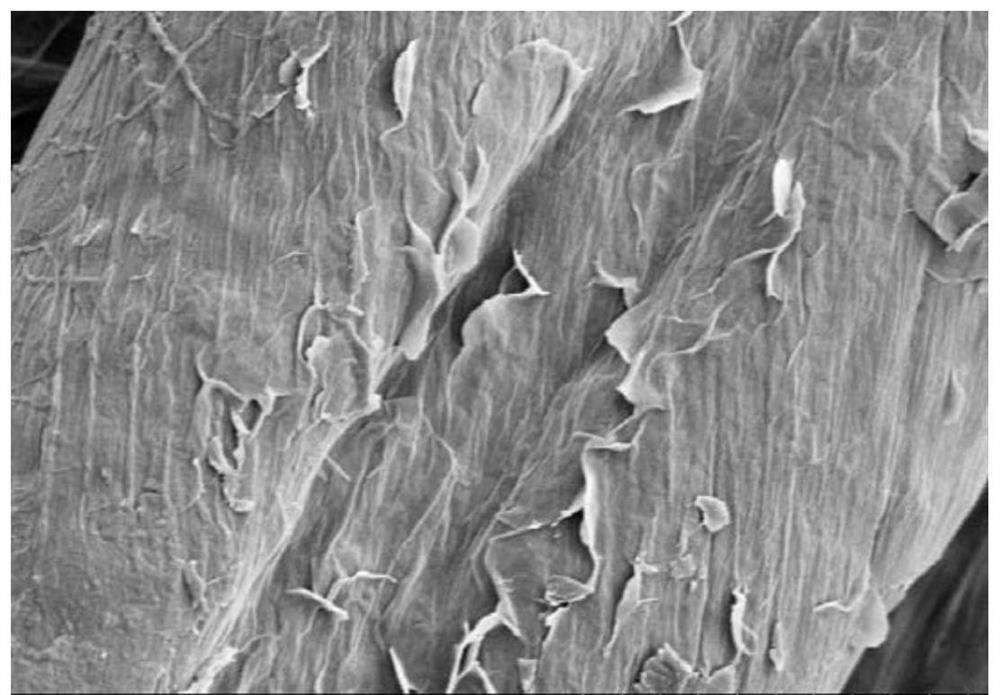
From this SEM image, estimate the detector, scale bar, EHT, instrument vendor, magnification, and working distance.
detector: SE(M)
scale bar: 5000 nm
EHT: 3 kV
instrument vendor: Hitachi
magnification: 10 K X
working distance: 10.3 mm